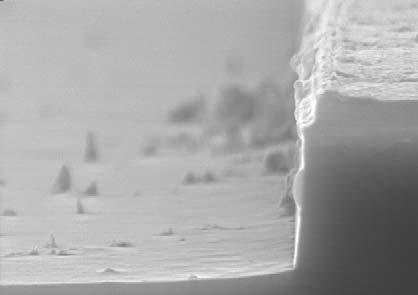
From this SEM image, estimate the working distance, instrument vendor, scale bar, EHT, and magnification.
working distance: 11.5 mm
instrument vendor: Hitachi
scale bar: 1000 nm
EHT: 15 kV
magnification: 30 K X